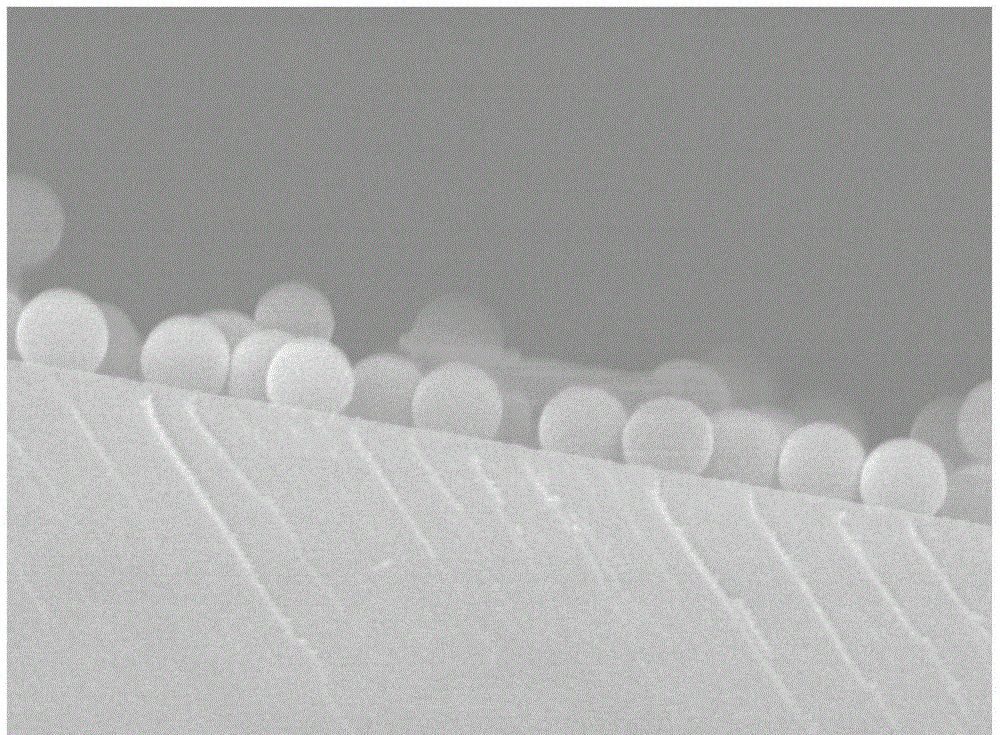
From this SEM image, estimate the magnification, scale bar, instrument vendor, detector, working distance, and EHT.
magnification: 30 K X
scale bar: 100 nm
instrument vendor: JEOL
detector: SEI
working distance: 7.4 mm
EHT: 10 kV